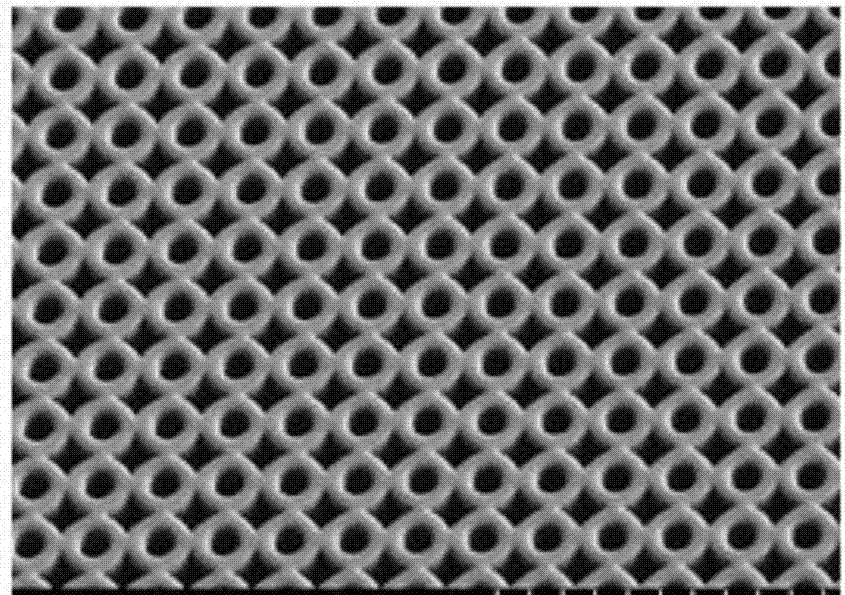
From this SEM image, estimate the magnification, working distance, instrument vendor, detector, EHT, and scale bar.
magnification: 0.5 K X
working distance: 10 mm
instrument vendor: Hitachi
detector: SE(L)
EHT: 1 kV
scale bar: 100000 nm